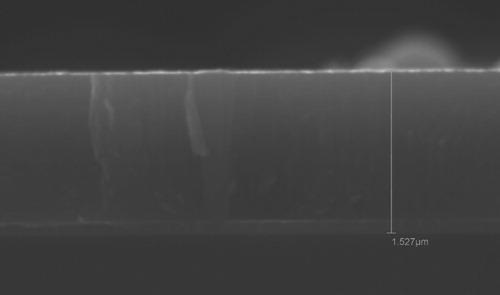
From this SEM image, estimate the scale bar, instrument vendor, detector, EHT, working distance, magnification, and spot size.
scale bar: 1000 nm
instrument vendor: JEOL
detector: SEI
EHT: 20 kV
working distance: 8 mm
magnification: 15 K X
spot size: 43